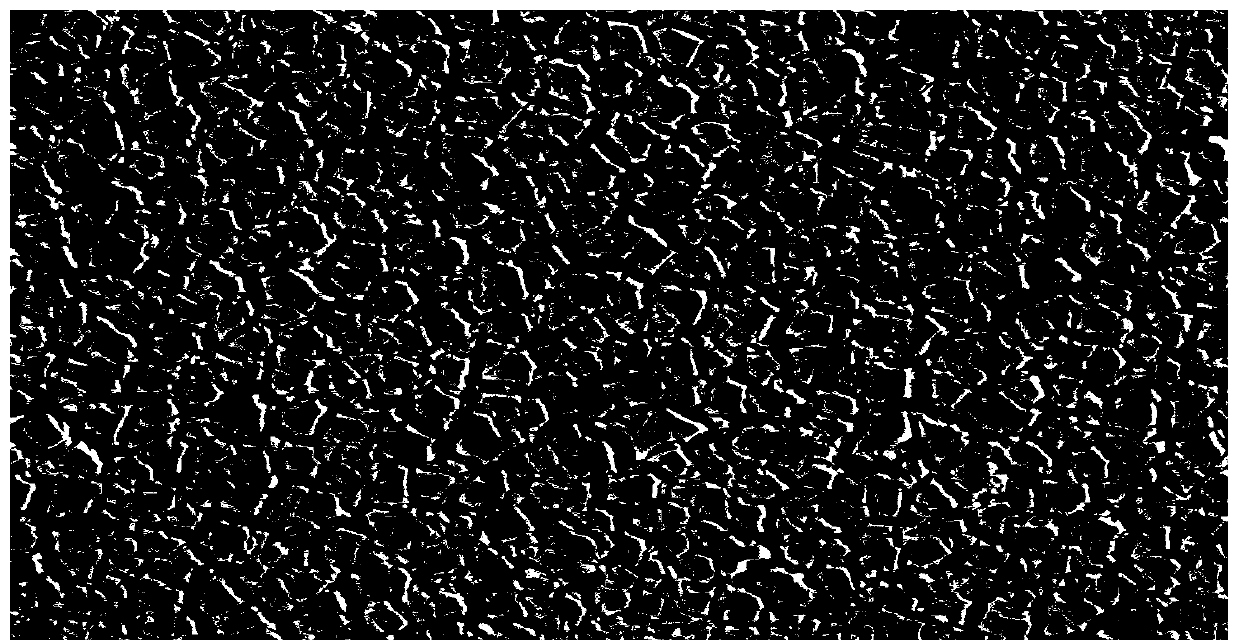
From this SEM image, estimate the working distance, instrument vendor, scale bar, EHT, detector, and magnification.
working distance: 10.1 mm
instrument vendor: Zeiss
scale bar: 10000 nm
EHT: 20 kV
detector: SE2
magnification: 0.5 K X